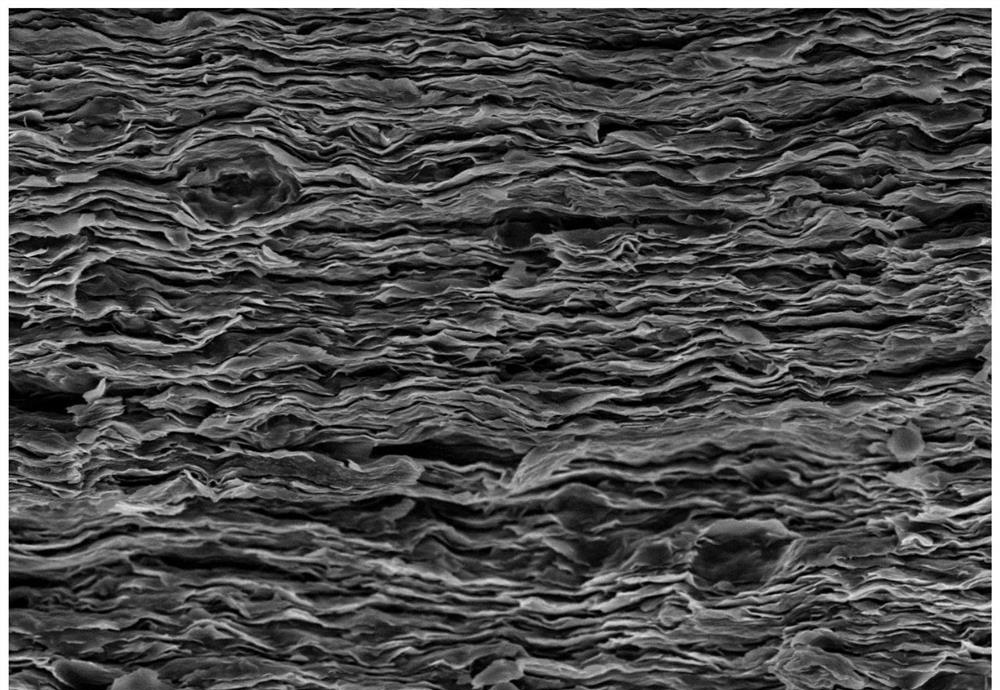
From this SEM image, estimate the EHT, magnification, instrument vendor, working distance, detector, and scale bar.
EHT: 5 kV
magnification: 5 K X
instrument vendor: Hitachi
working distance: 9.2 mm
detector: SE(M,LA0)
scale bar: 10000 nm